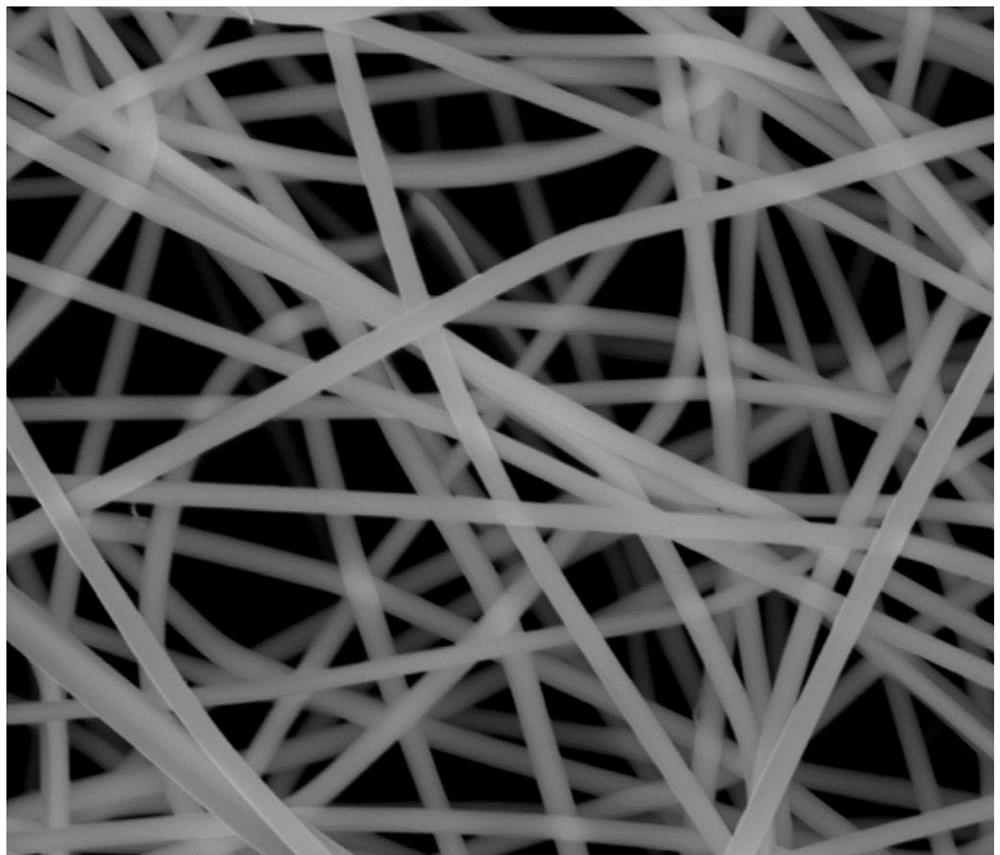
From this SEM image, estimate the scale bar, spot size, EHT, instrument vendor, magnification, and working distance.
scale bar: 5000 nm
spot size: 4.5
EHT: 14.98 kV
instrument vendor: FEI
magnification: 20 K X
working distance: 14.8 mm